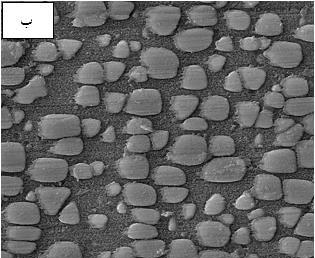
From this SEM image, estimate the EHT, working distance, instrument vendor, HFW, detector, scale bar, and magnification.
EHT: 15 kV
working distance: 11.6 mm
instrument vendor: Tescan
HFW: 5.93 µm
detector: SE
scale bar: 1000 nm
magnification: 35 K X